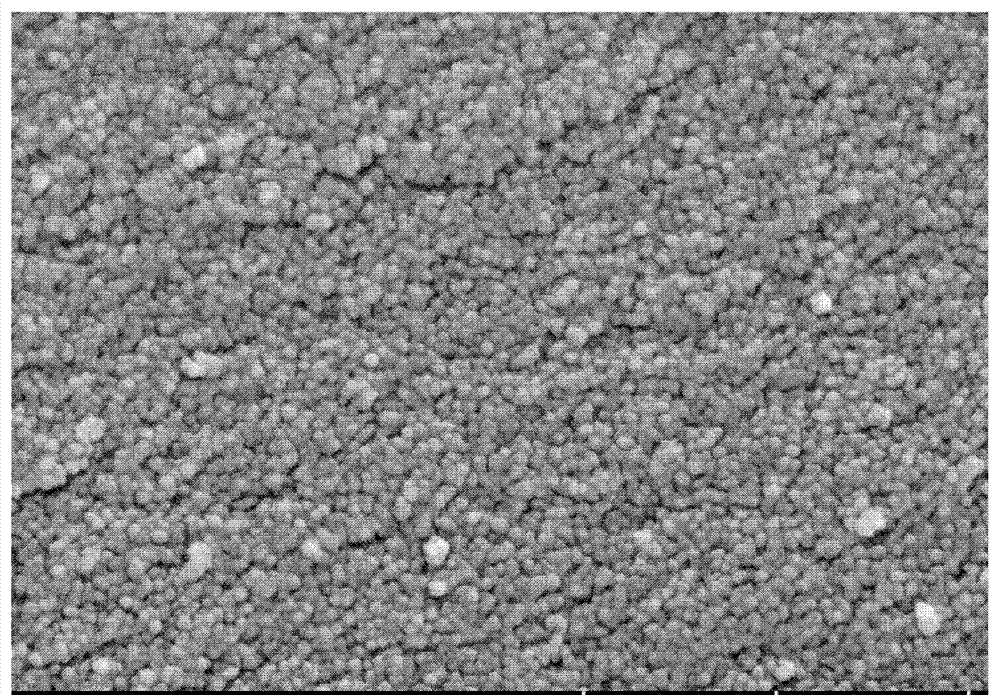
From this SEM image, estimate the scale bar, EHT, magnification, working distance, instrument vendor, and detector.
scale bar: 500 nm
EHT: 5 kV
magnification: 100 K X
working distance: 5.7 mm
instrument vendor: Hitachi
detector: SE(U)